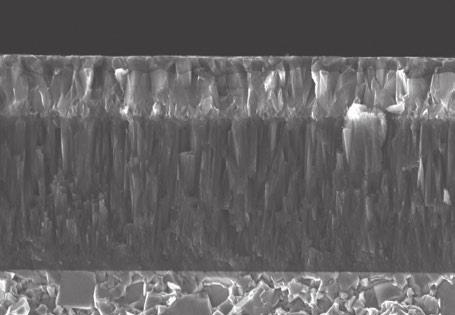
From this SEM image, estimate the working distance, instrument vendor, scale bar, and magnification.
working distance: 11.8 mm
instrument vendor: JEOL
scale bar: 2500 nm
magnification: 4 K X